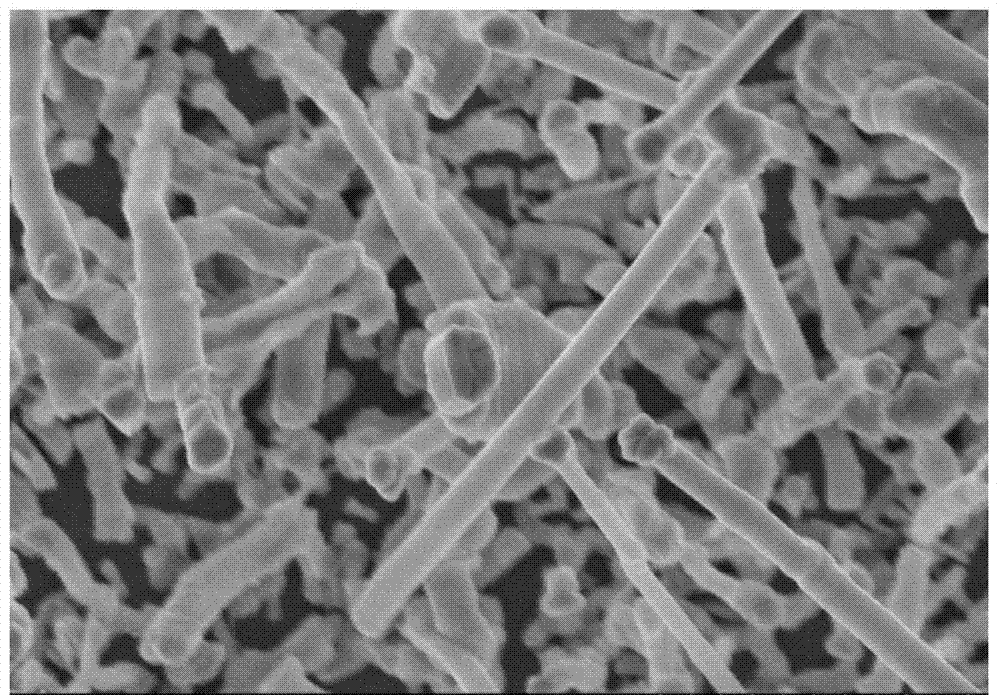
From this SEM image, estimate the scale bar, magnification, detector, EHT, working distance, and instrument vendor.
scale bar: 2000 nm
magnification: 20 K X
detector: SE(M)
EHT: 5 kV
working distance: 6.6 mm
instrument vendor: Hitachi